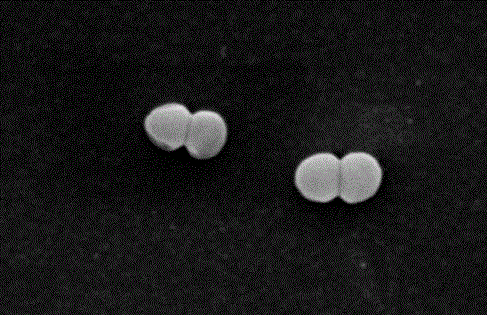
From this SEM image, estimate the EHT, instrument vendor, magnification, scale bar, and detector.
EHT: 10 kV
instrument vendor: JEOL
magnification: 20 K X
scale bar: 1000 nm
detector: SEI